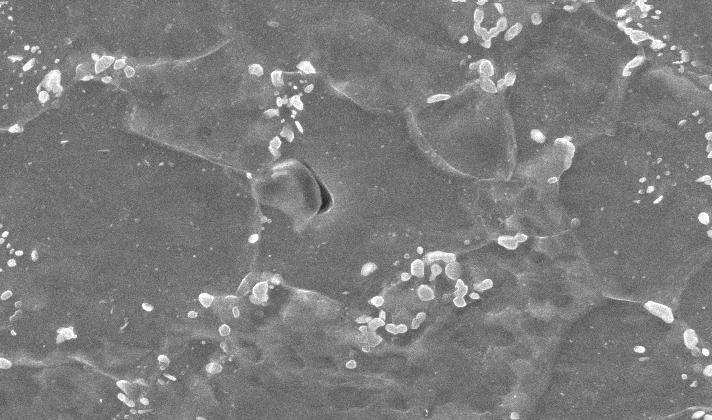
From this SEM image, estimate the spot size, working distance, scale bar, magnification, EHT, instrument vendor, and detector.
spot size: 3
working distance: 10 mm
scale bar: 5000 nm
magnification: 10 K X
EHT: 25 kV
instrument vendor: FEI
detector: SE